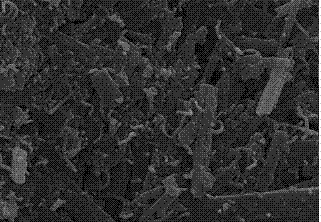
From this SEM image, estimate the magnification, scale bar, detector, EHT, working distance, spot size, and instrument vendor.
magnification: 20 K X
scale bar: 1000 nm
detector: SE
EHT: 20 kV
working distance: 5.1 mm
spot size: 3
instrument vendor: FEI